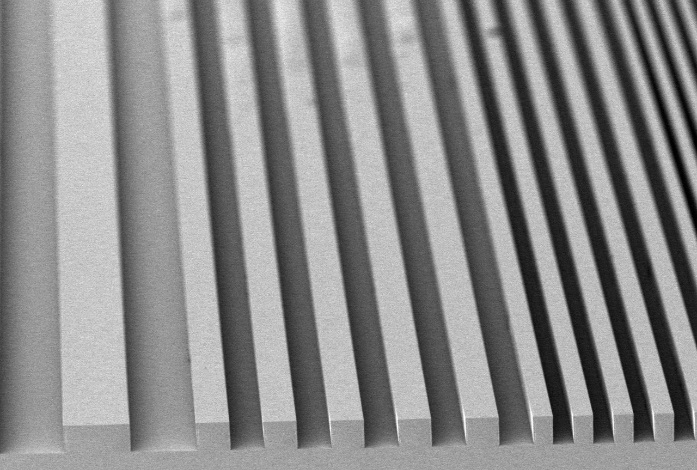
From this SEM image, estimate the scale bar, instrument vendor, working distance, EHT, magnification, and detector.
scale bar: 250000 nm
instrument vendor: Hitachi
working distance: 5 mm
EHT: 3 kV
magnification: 0.12 K X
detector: SE